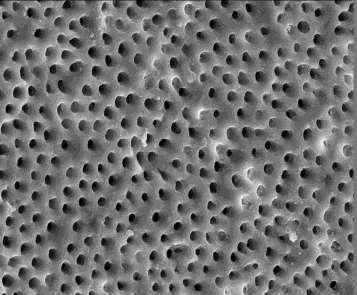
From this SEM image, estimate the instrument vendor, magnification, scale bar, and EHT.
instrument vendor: JEOL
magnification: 1 K X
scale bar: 10000 nm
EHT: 20 kV